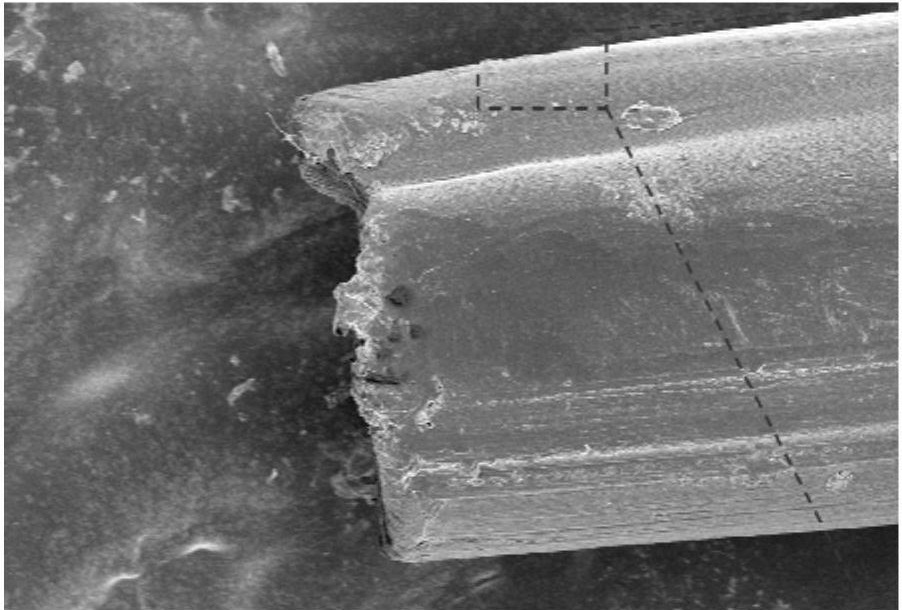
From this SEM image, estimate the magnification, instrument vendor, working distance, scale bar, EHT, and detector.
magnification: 0.191 K X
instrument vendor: Zeiss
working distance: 3.4 mm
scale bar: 20000 nm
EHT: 5 kV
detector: InLens